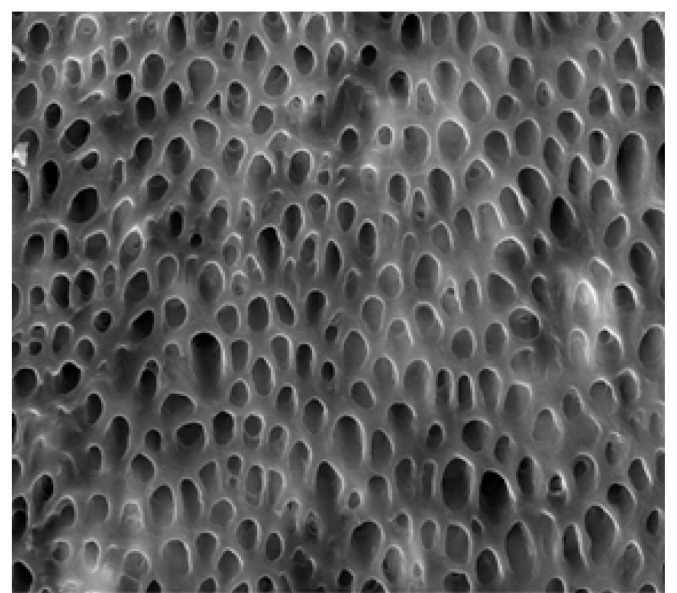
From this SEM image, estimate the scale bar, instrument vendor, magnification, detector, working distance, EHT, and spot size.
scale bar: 10000 nm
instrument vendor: FEI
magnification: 5 K X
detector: ETD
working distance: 4.6 mm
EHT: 15 kV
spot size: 3